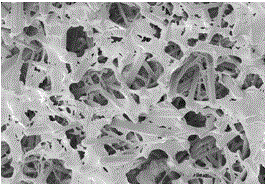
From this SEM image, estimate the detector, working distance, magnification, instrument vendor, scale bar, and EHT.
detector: SE(M,LA0)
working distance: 10.7 mm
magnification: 5 K X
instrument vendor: Hitachi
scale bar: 10000 nm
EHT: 5 kV